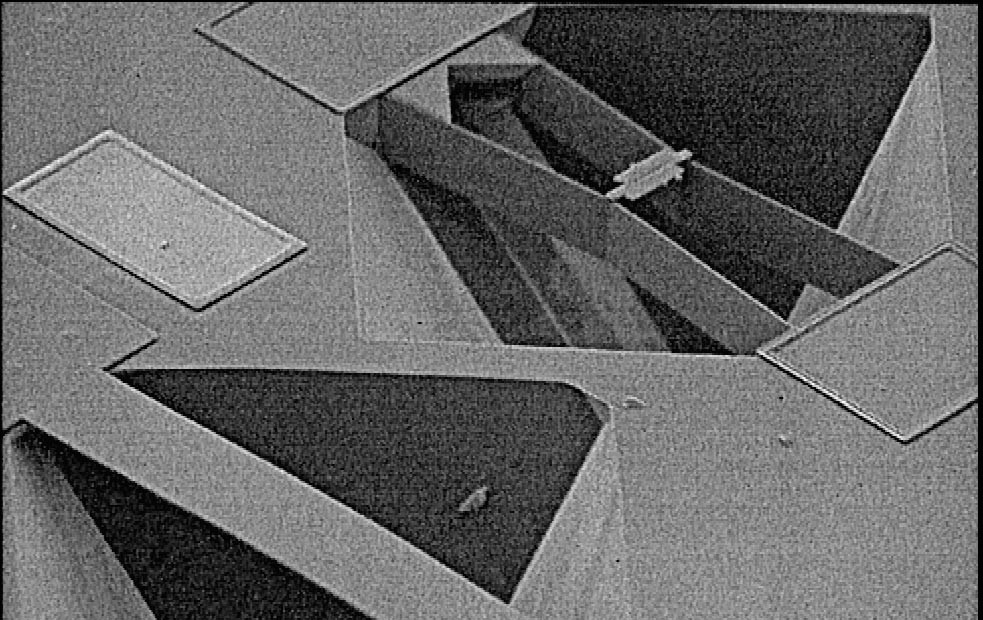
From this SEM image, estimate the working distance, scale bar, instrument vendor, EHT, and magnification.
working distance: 39 mm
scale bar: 100000 nm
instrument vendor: JEOL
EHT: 10 kV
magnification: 0.14 K X